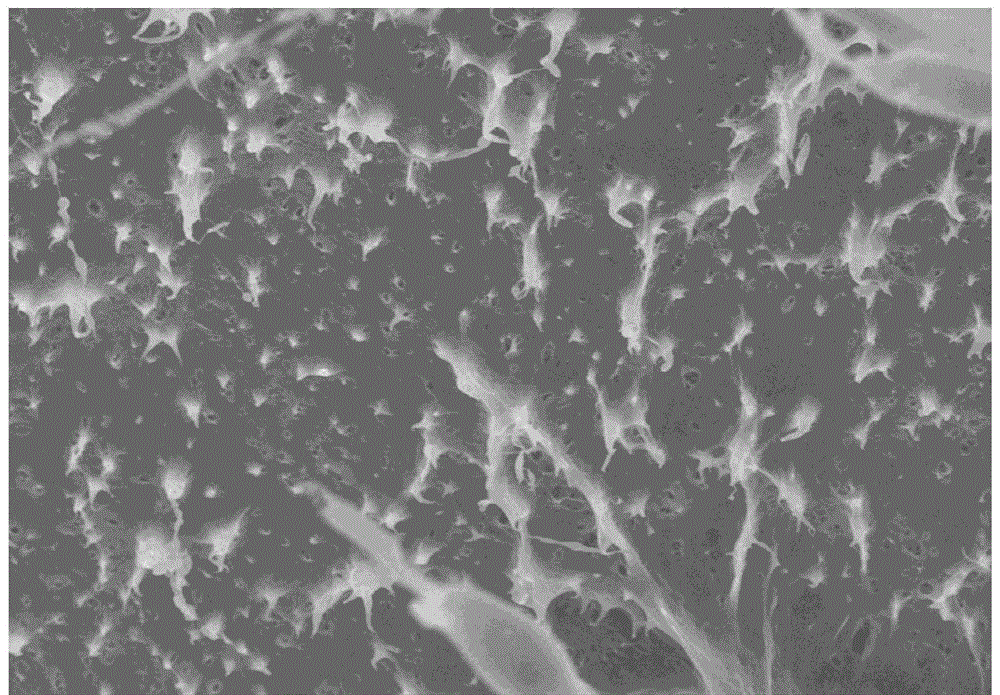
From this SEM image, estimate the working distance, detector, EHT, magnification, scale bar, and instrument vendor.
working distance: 12.3 mm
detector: SE(M)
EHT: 10 kV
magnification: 3 K X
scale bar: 10000 nm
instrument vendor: Hitachi